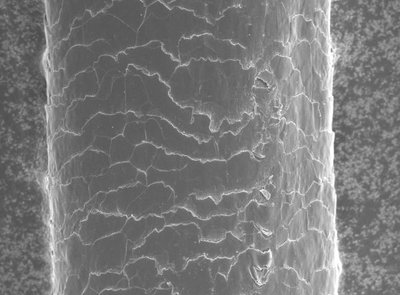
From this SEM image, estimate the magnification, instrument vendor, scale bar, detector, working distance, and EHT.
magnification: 1 K X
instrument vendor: JEOL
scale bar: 10000 nm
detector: SEI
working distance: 8 mm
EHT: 5 kV